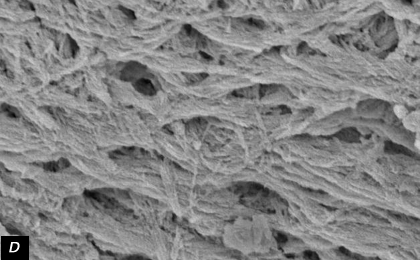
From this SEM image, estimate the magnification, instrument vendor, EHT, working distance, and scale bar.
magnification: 10 K X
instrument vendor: other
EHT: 5 kV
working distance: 25 mm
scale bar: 3000 nm